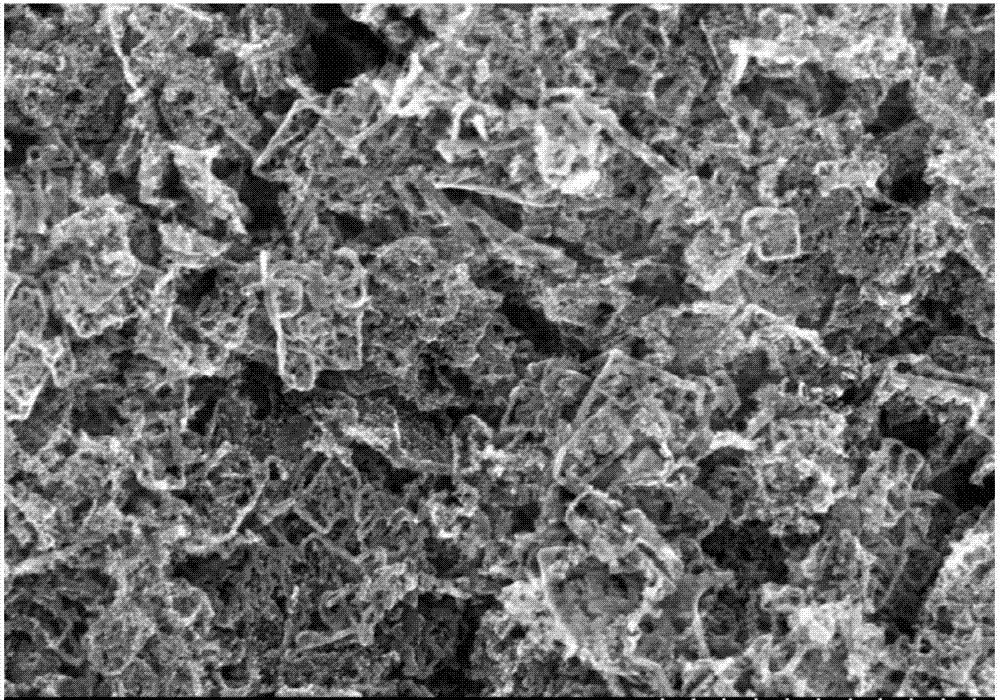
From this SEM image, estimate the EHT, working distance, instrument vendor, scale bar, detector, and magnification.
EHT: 20 kV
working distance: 8.6 mm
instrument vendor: Hitachi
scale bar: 2000 nm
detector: SE(M)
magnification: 20 K X